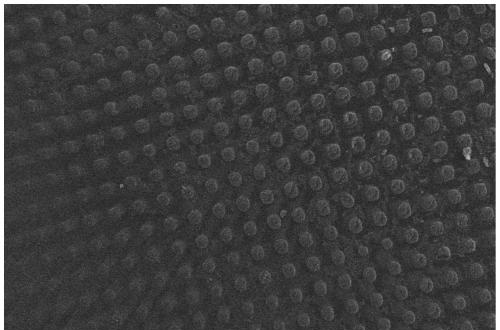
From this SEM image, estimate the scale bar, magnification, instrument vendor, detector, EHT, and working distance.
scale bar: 200000 nm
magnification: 0.095 K X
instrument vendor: JEOL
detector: SEI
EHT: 20 kV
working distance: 10 mm